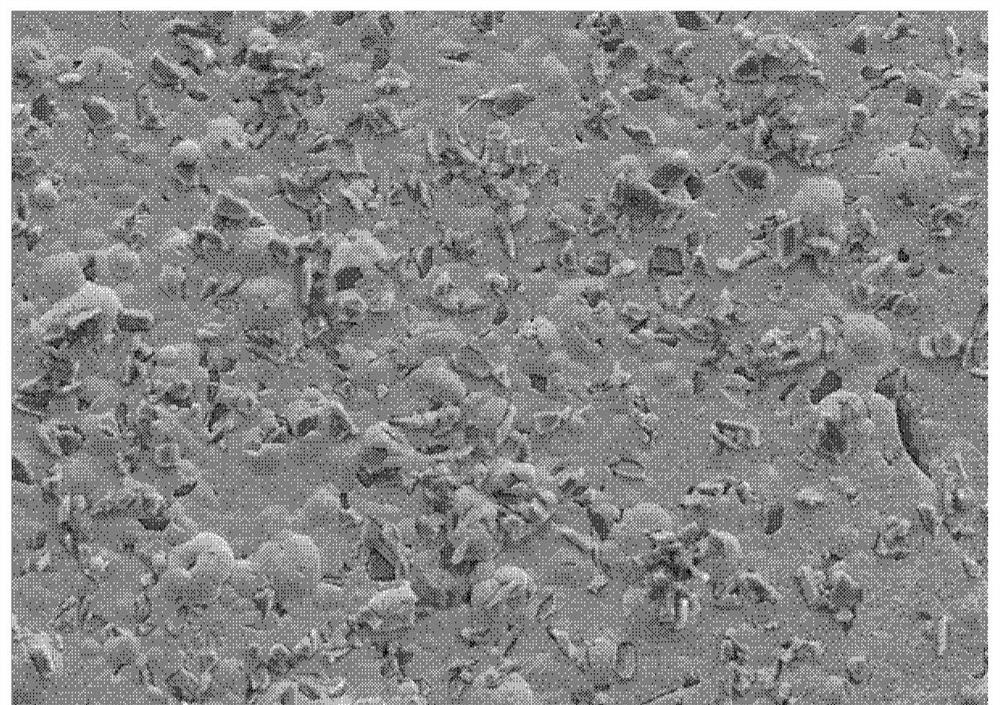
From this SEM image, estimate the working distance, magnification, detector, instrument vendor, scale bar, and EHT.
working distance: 11.9 mm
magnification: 0.5 K X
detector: SE2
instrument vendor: Zeiss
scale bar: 10000 nm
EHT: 15 kV